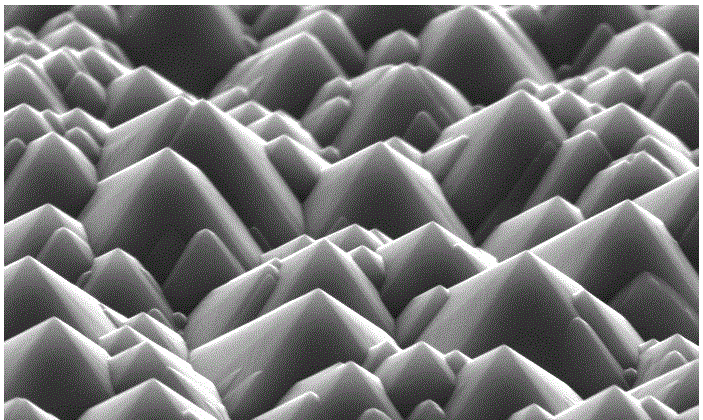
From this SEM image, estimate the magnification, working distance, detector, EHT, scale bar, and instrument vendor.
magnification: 5 K X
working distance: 9.4 mm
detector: SE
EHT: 20 kV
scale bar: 10000 nm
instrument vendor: FEI